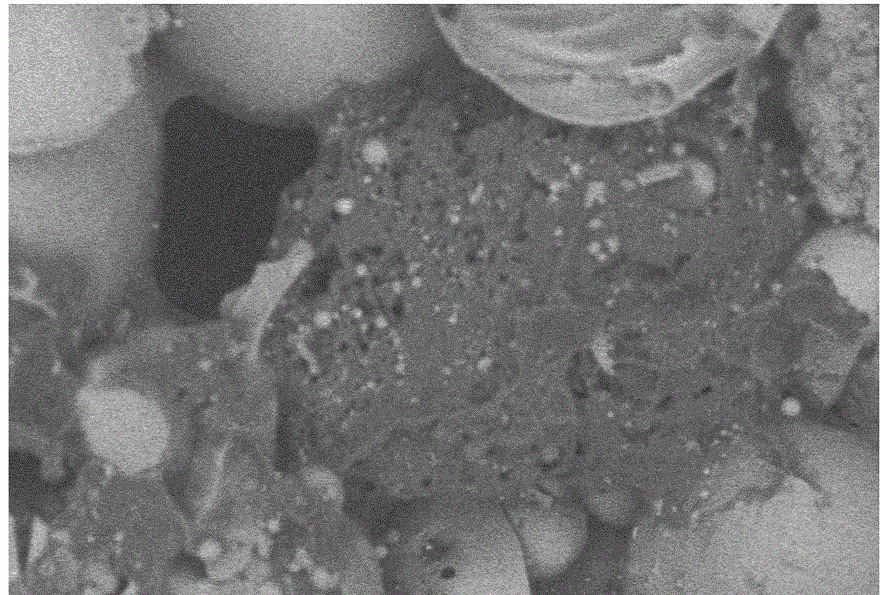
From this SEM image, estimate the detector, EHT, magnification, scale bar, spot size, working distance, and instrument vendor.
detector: BEC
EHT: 10 kV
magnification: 0.5 K X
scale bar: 50000 nm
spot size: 42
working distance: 10 mm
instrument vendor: JEOL